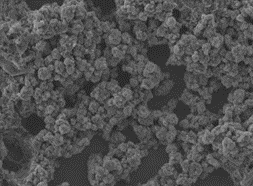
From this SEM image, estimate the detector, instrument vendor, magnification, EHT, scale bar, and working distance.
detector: LED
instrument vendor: JEOL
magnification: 3 K X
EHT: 5 kV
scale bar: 1000 nm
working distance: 10 mm